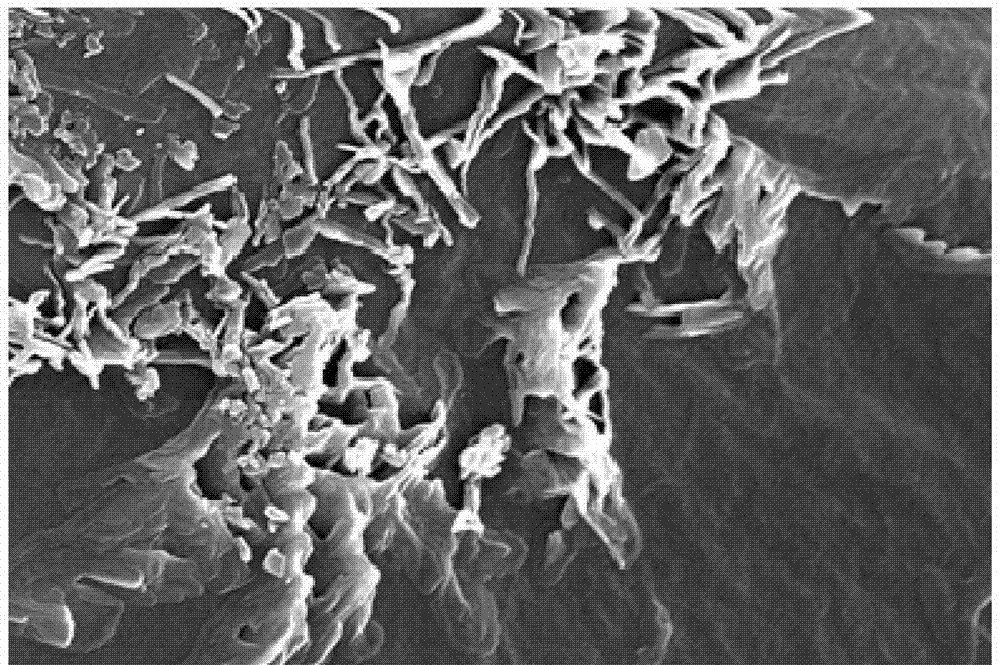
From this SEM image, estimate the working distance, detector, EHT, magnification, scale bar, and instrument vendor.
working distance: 5.8 mm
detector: InLens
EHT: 15 kV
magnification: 24.45 K X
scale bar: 200 nm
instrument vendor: Zeiss